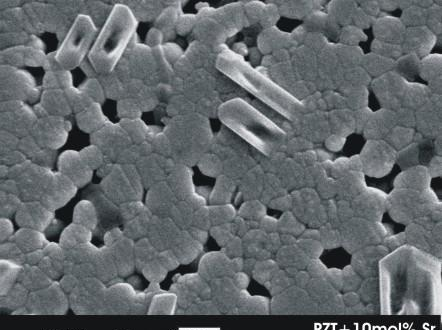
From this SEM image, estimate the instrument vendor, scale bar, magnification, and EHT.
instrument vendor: JEOL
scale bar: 2000 nm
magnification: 5 K X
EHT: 20 kV